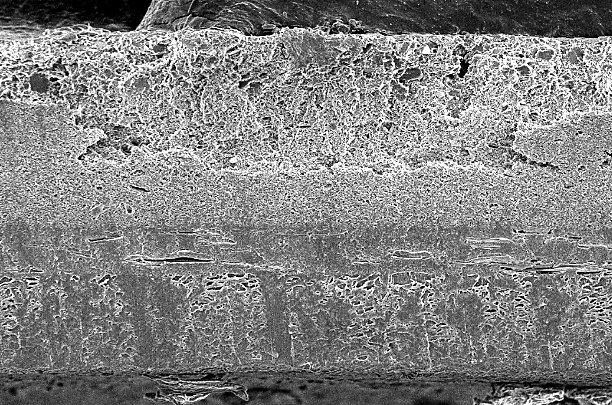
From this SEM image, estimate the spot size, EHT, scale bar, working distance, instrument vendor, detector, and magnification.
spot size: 13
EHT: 5 kV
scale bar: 10000 nm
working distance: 12.9 mm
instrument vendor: other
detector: SE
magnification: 1 K X